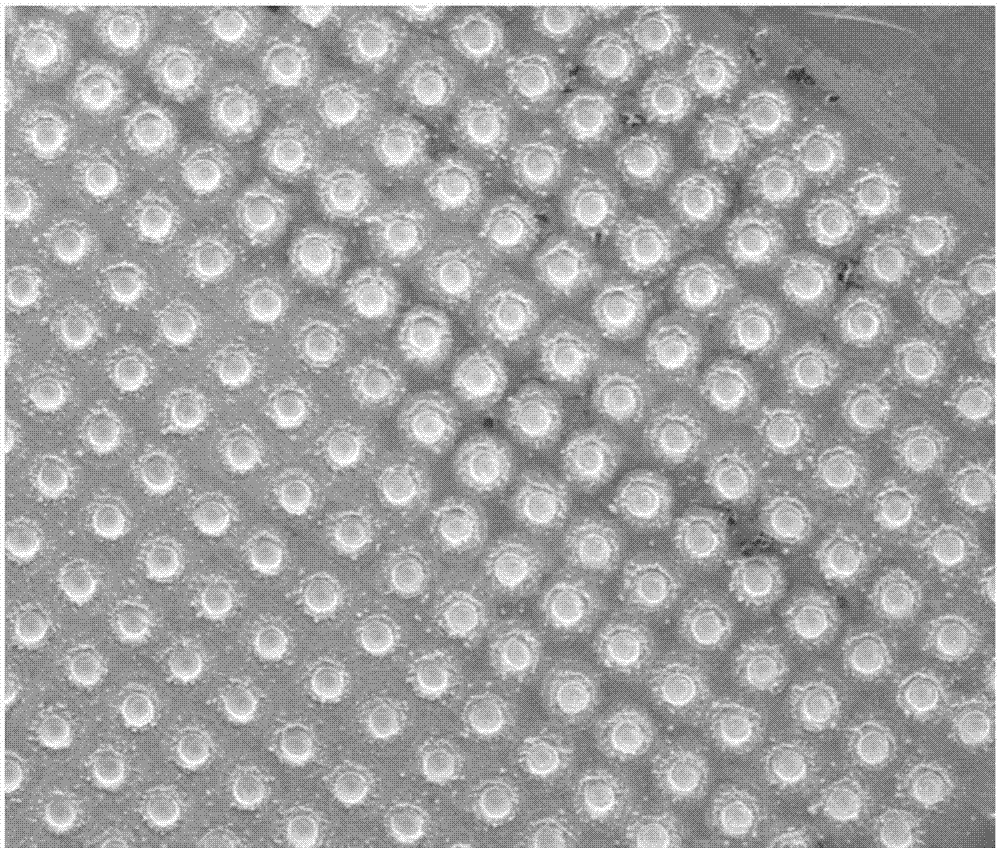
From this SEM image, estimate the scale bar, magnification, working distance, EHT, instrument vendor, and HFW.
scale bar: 500000 nm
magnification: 0.2 K X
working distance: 16.3 mm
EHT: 15 kV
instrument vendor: FEI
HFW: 1490 µm